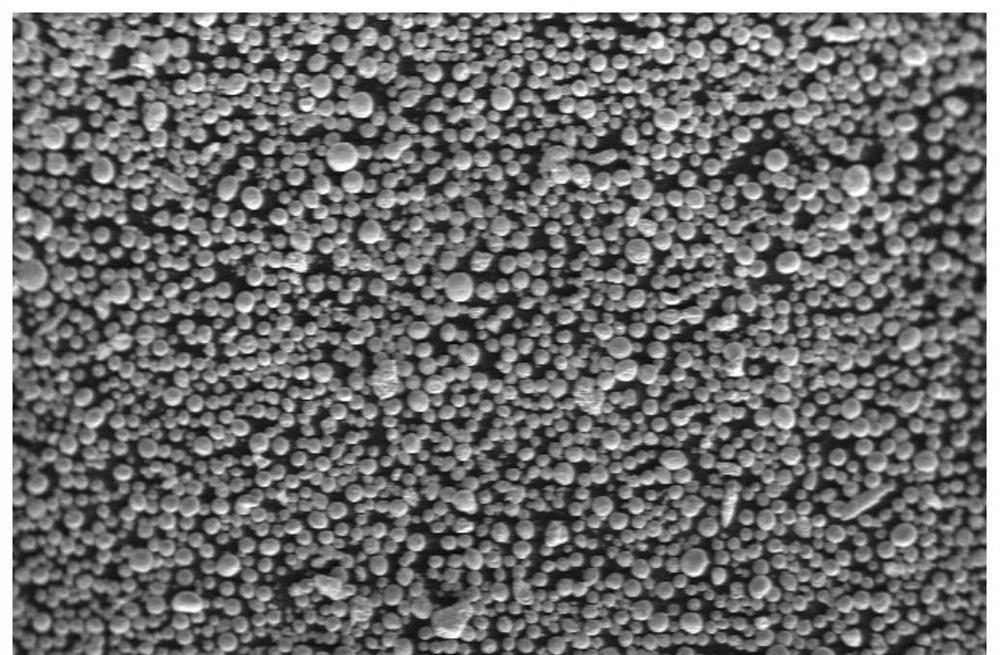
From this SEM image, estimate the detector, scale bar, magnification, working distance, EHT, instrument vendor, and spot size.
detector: SE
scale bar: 500000 nm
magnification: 0.05 K X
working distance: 9.8 mm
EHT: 5 kV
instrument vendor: FEI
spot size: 3